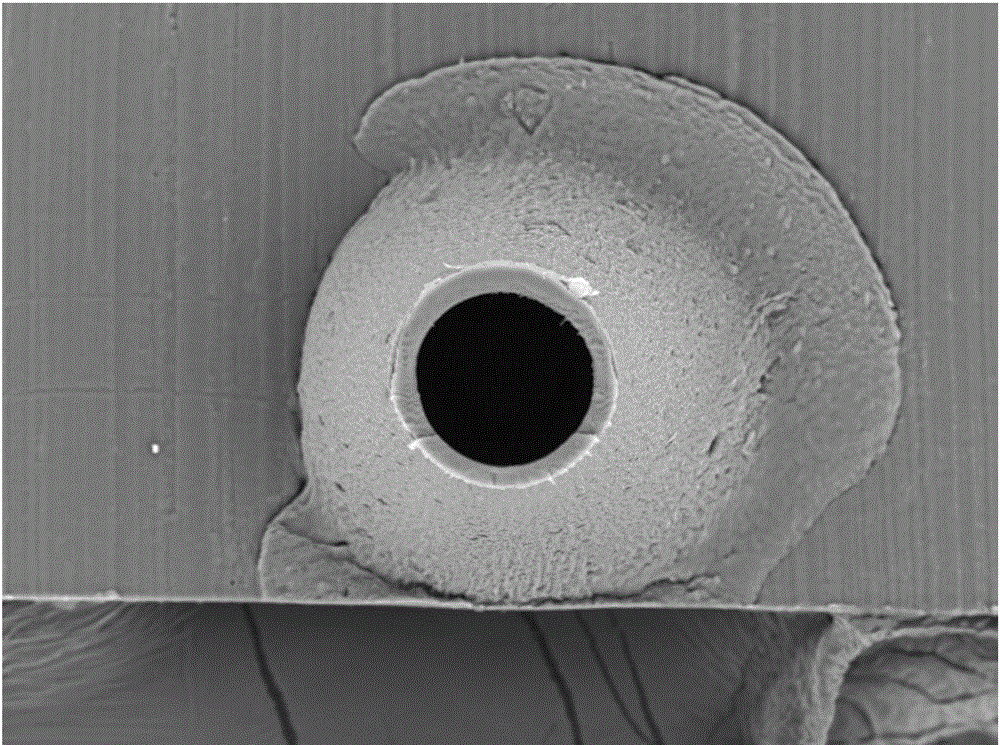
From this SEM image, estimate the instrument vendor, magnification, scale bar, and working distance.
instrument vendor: Hitachi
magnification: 0.5 K X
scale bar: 200000 nm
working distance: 4.5 mm